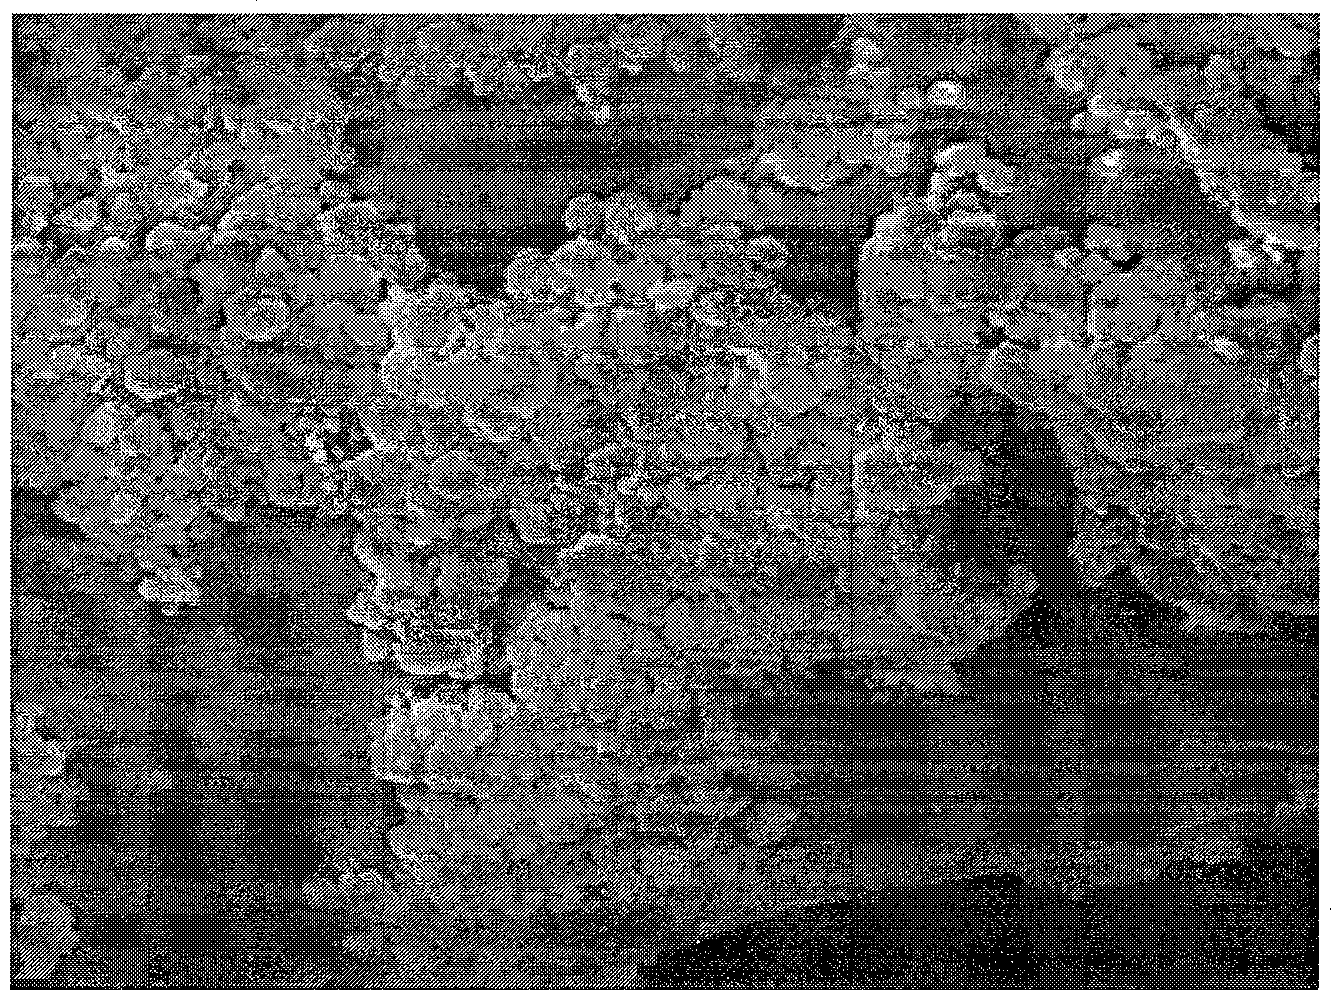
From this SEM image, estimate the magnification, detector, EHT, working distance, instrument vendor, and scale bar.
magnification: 3 K X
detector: SEI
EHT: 10 kV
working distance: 9.6 mm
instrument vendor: JEOL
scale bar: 1000 nm